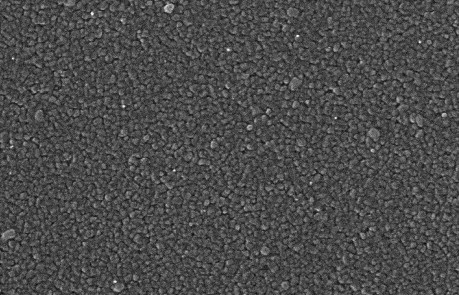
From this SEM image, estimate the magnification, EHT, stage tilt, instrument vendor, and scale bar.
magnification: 50 K X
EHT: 30 kV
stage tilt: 0°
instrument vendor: JEOL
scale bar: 600 nm